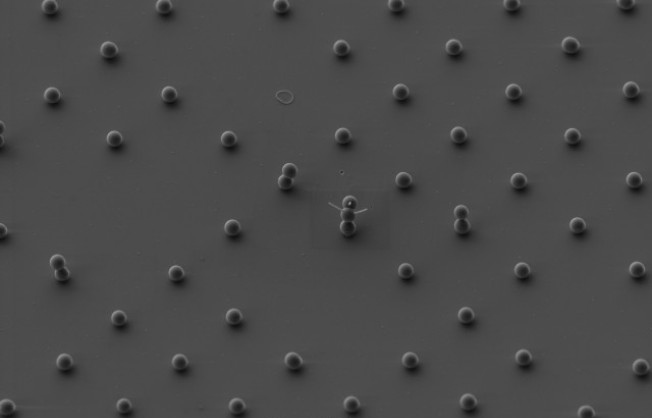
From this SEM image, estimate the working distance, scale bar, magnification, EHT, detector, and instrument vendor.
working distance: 4.4 mm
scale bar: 2000 nm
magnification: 3 K X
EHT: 3 kV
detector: SE2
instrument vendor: Zeiss